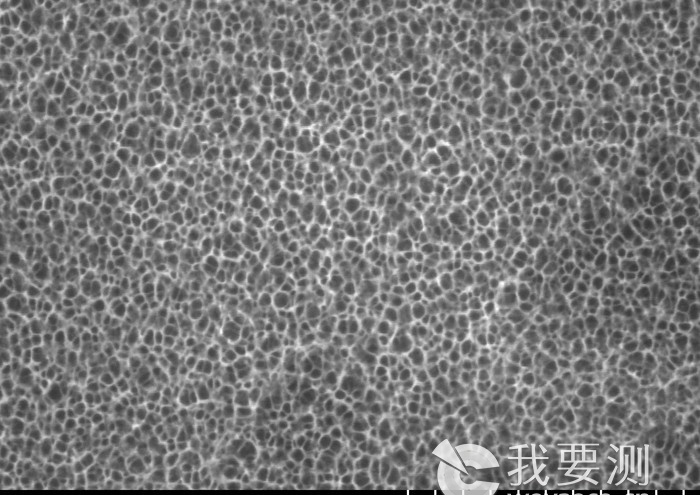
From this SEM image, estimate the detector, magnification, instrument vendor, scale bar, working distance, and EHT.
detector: SE(UL)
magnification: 100 K X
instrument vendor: Hitachi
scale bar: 500 nm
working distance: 8.2 mm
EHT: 10 kV